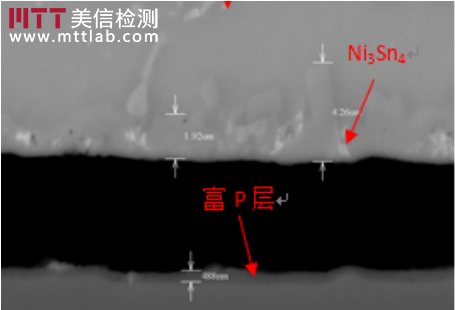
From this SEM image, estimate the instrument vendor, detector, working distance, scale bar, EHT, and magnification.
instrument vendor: Hitachi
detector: BSE3D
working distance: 10.2 mm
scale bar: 5000 nm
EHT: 25 kV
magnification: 6.5 K X